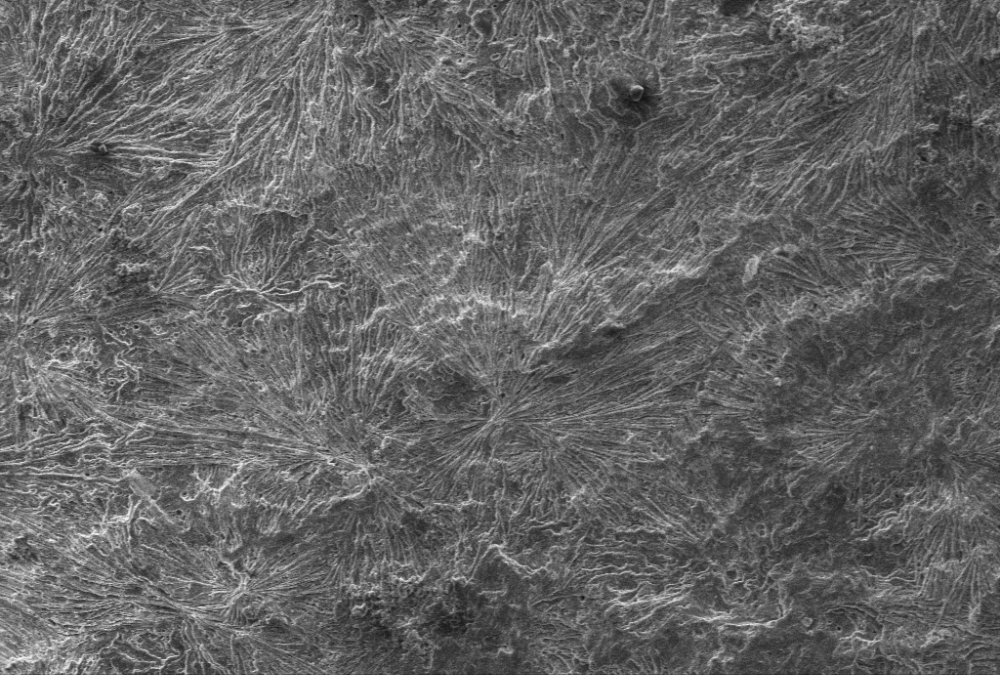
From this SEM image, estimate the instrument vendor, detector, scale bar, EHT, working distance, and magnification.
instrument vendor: Zeiss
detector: SE1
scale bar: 100000 nm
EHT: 20 kV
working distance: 10 mm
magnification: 0.1 K X